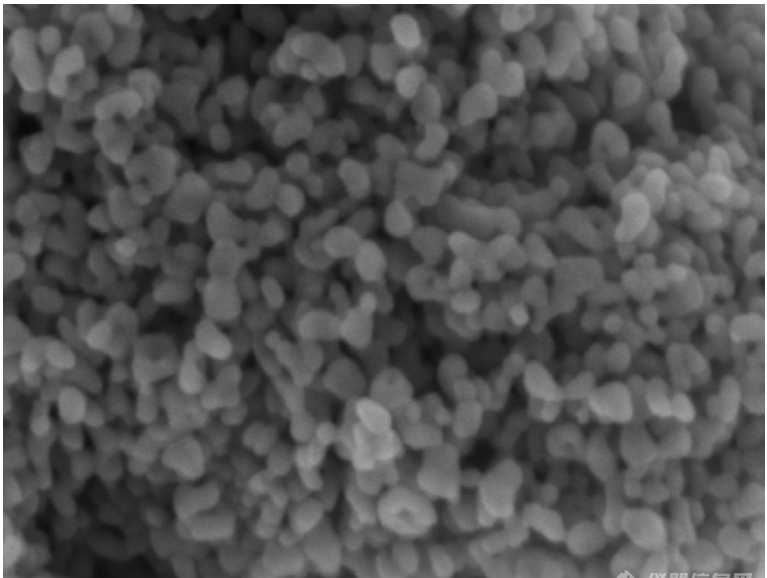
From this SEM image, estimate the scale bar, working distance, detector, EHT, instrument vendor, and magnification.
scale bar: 100 nm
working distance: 4.8 mm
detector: InLens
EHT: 3 kV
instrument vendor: Zeiss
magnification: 200 K X